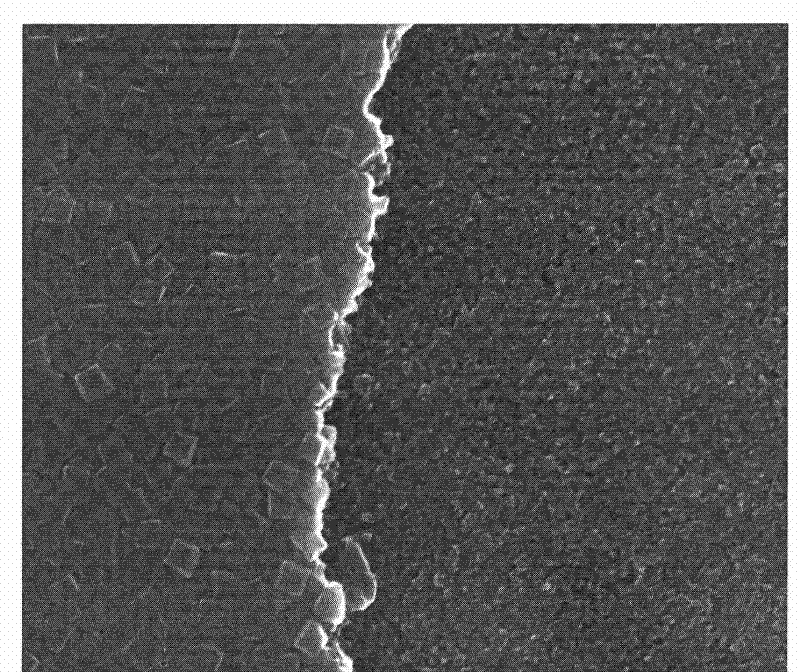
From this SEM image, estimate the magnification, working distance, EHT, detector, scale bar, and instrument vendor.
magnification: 129.524 K X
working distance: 5 mm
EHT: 15 kV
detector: TLD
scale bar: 500 nm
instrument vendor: FEI